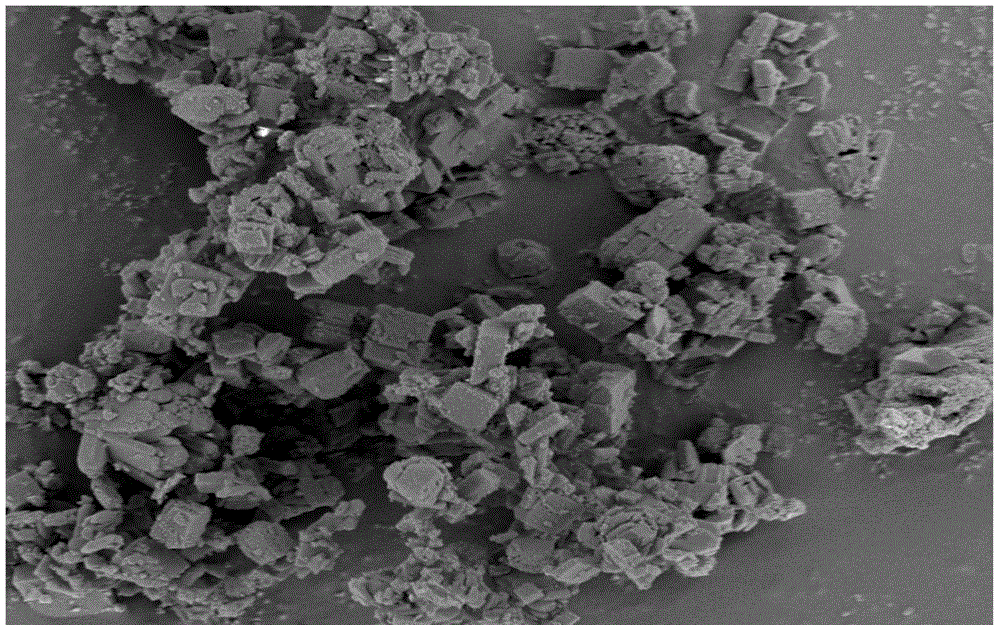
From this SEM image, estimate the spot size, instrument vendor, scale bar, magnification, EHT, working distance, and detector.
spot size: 3.5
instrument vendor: FEI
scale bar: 5000 nm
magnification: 20 K X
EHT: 3 kV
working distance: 4.7 mm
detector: TLD